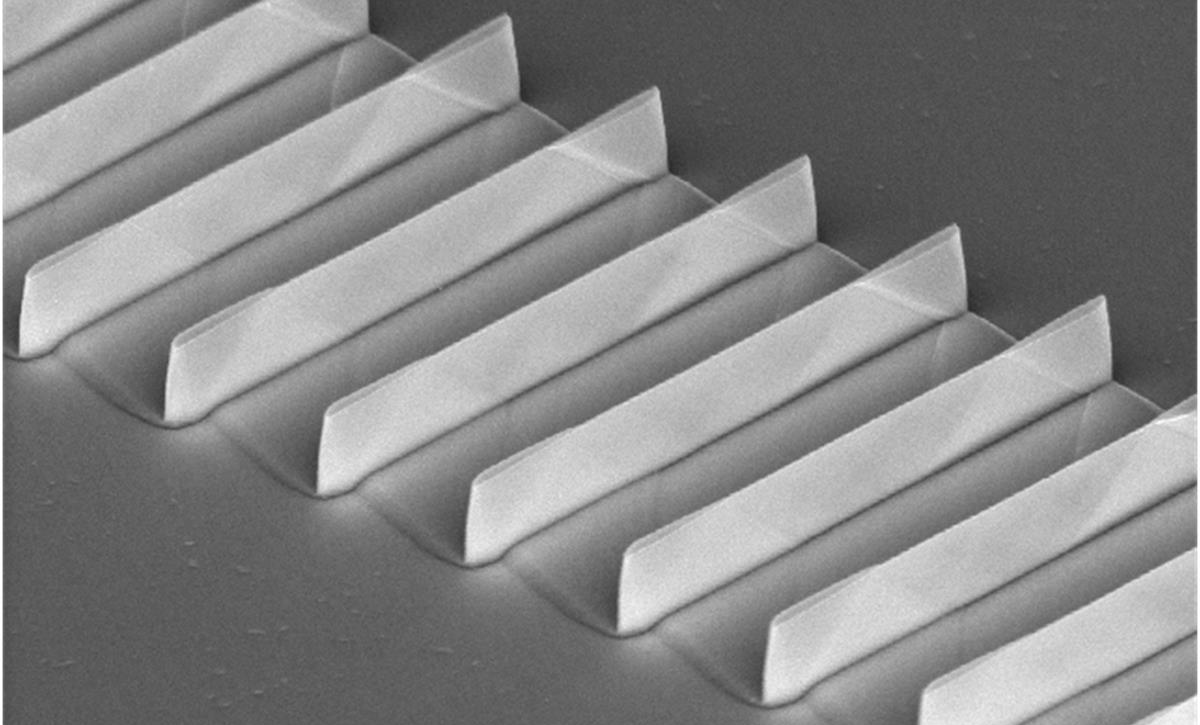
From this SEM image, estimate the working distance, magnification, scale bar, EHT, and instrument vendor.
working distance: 14.1 mm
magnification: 15 K X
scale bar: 3000 nm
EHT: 5 kV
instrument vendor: Hitachi